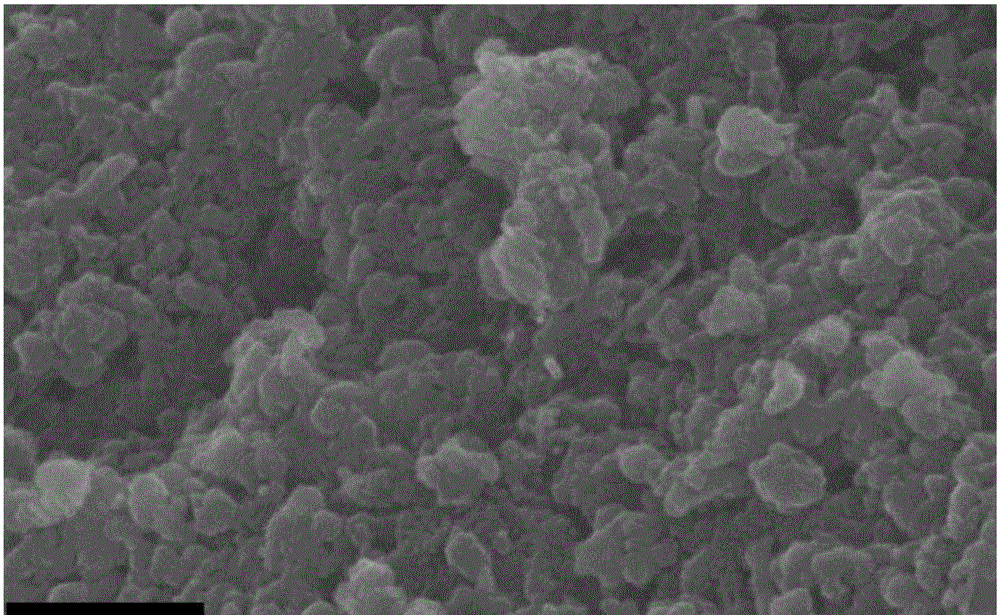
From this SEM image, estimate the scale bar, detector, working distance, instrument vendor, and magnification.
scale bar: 500 nm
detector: TLD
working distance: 5.1 mm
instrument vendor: FEI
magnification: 40 K X